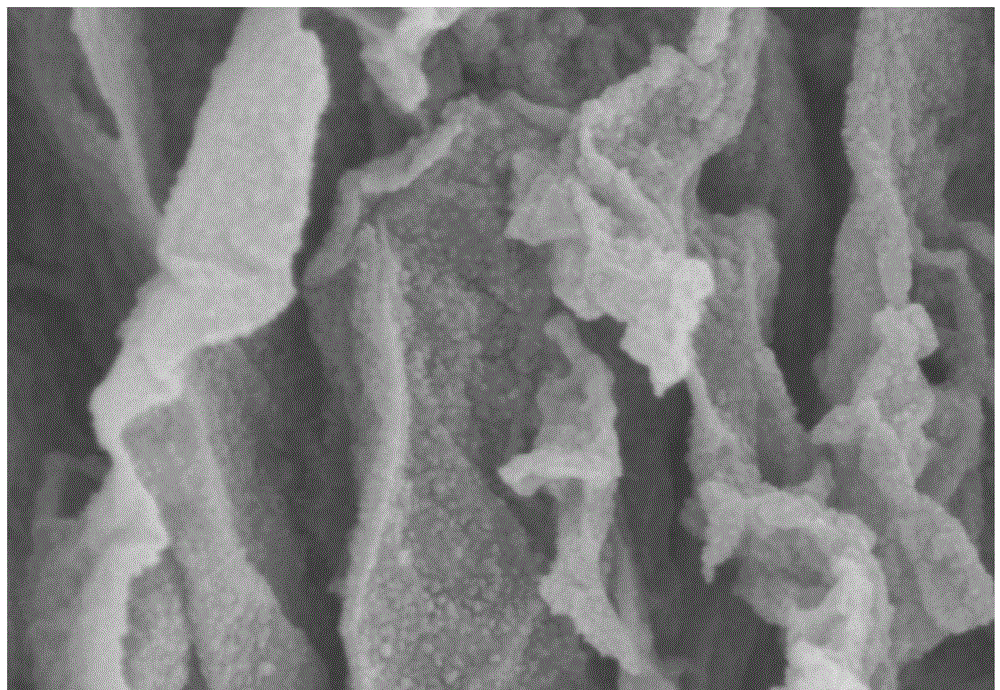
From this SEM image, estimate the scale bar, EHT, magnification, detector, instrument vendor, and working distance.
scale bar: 500 nm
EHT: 3 kV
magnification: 100 K X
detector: SE(M)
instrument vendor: Hitachi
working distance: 8.9 mm